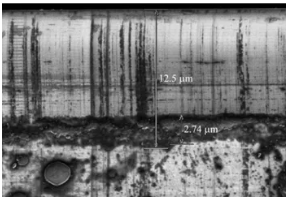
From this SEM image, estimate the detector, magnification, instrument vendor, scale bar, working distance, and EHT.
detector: SE(UL)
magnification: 5 K X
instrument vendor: Hitachi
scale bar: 10000 nm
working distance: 12.5 mm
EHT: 1 kV